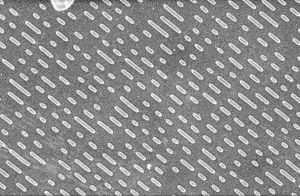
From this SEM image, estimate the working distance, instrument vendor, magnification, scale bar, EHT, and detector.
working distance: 5 mm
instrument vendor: Hitachi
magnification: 3 K X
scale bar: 10000 nm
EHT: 5 kV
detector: SE(M)